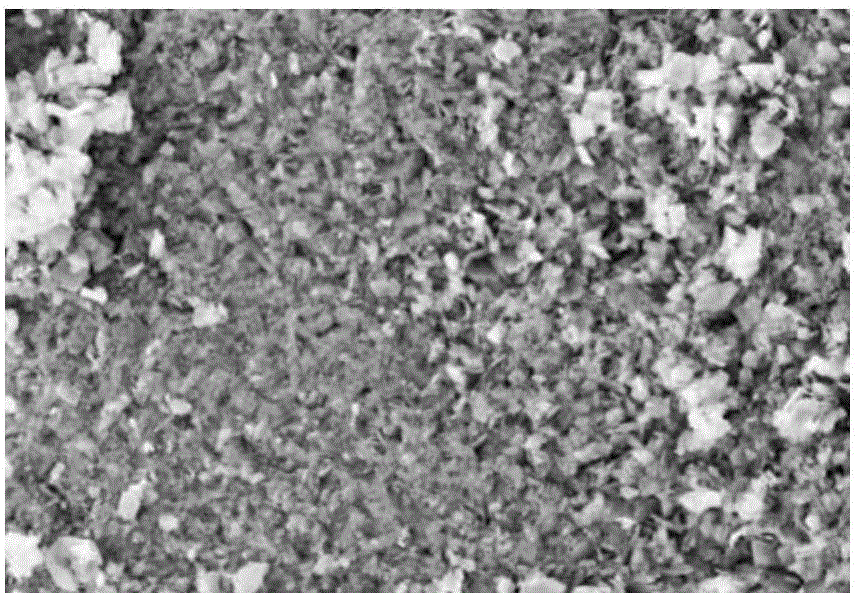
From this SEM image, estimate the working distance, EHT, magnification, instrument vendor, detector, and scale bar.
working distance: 7.1 mm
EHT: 3 kV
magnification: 20 K X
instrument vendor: Zeiss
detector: SE2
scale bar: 100 nm